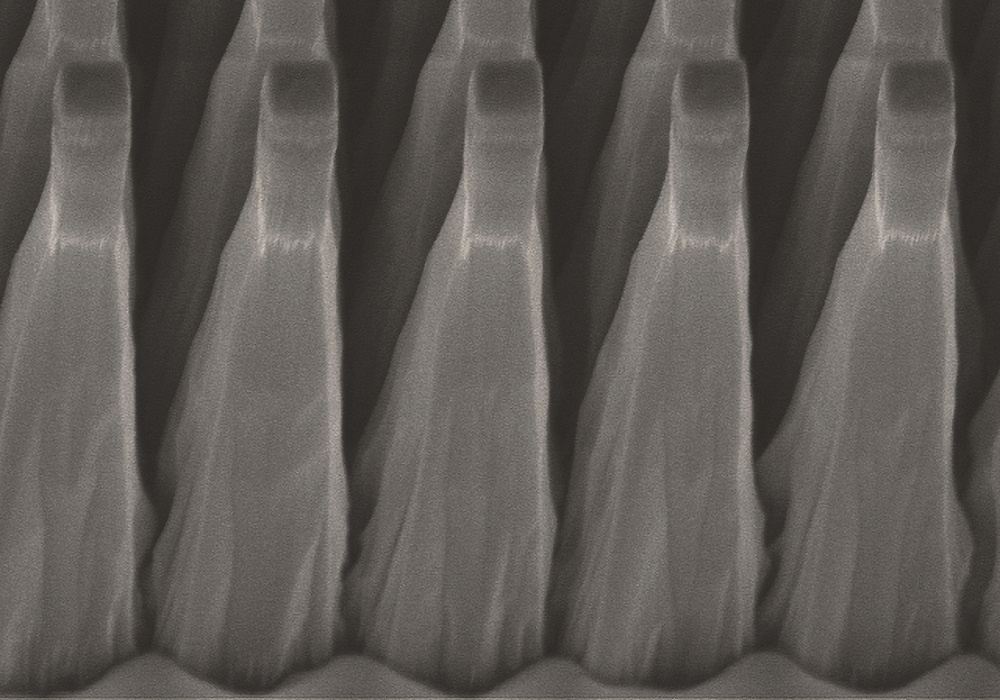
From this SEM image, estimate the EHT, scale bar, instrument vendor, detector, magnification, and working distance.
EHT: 5 kV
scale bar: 4000 nm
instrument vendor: Hitachi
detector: SE(U)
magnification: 12 K X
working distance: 11.7 mm